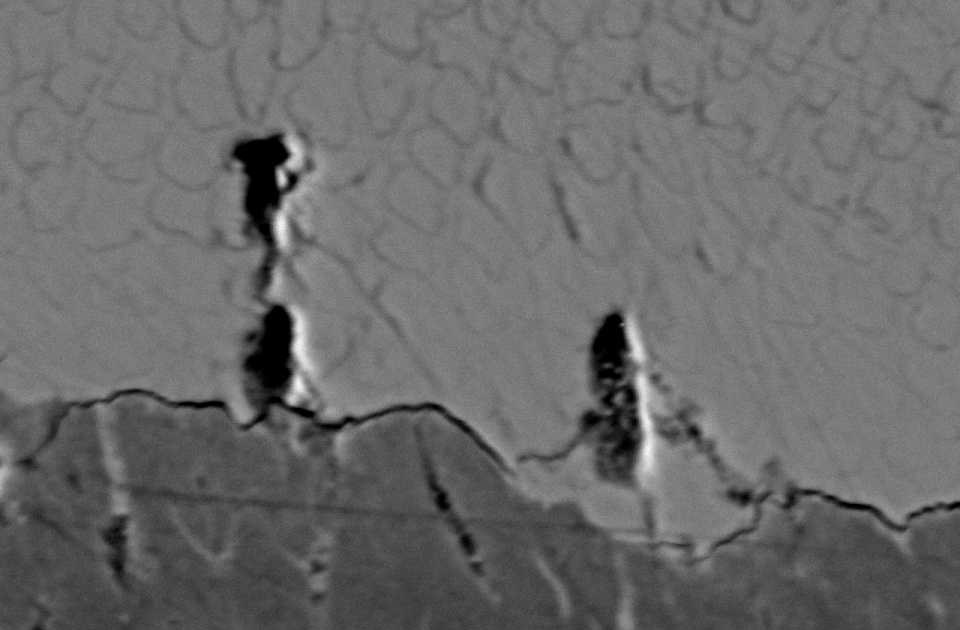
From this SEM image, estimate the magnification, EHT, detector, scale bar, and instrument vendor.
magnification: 1.7 K X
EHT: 15 kV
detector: BES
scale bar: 10000 nm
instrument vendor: JEOL